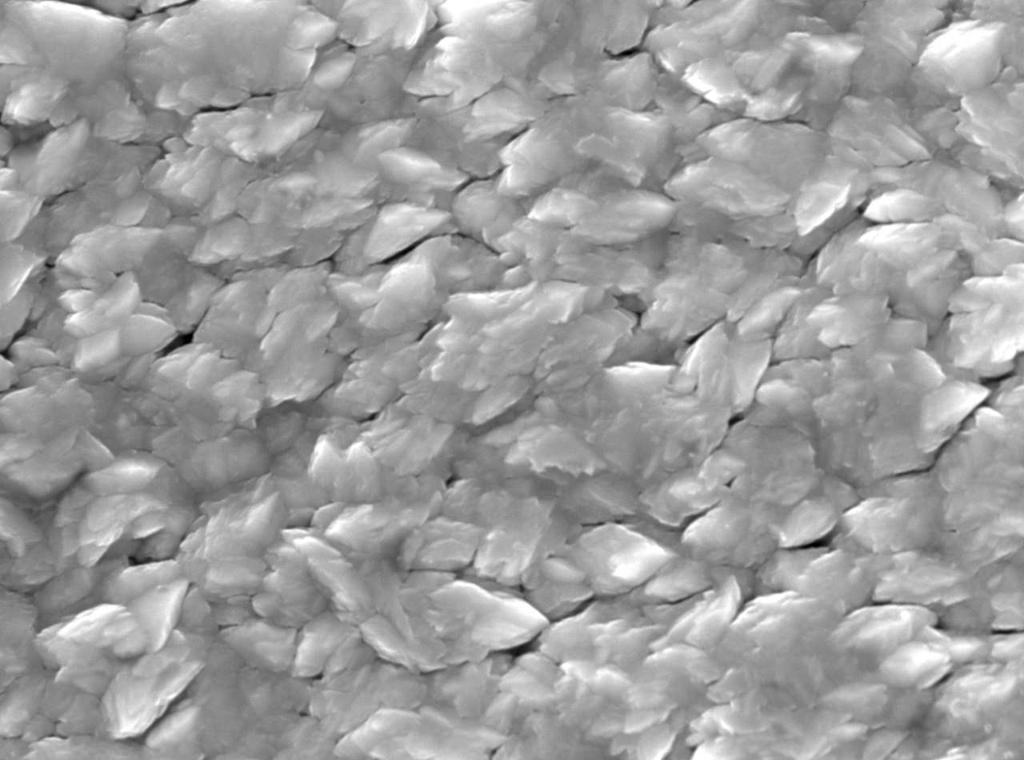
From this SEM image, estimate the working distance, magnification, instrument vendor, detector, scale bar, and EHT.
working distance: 11.1 mm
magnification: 10 K X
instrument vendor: JEOL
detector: SEI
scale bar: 1000 nm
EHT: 15 kV